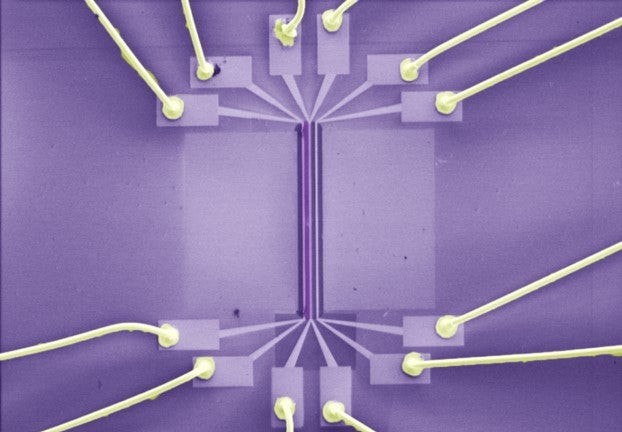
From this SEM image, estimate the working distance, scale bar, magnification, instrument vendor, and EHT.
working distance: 10.1 mm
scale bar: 1000000 nm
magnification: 0.04 K X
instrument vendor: Hitachi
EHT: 1 kV